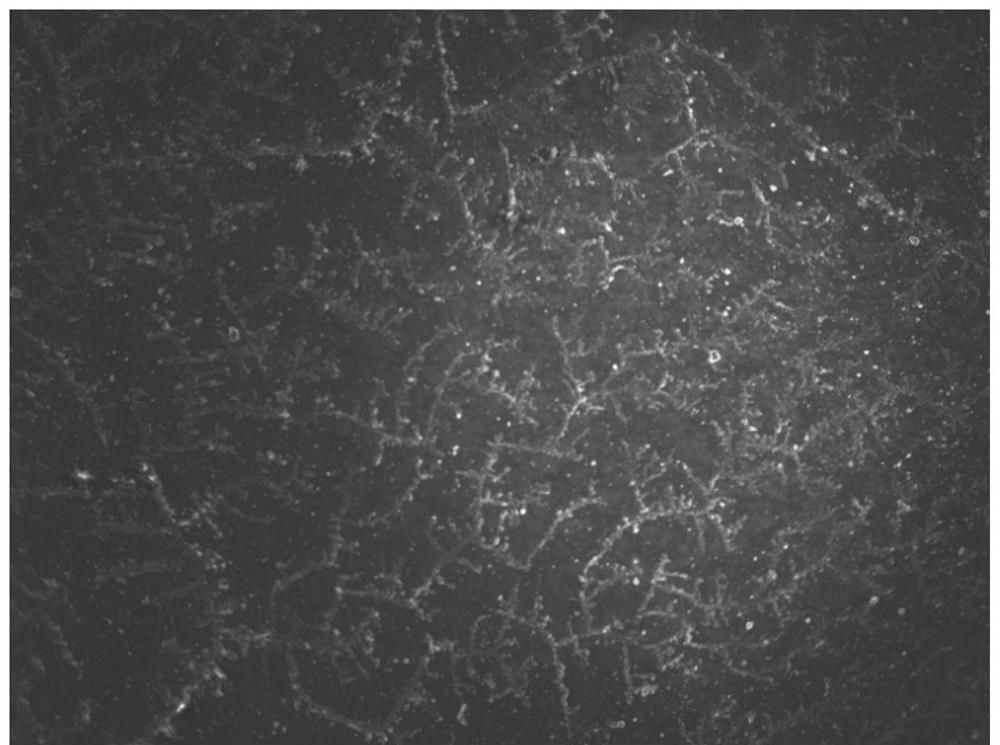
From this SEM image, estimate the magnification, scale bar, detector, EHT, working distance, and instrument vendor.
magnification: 0.25 K X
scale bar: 100000 nm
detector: SEI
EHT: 10 kV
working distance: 8 mm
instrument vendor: JEOL